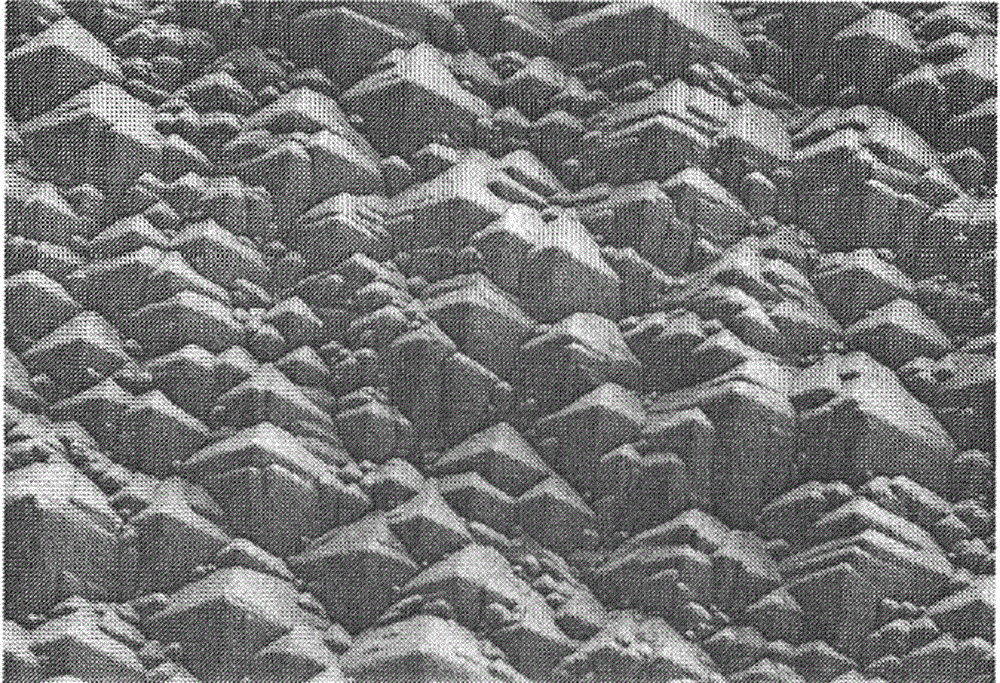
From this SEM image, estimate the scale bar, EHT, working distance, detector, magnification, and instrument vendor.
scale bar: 20000 nm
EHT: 5 kV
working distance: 9.3 mm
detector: SE(M)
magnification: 2 K X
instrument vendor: Hitachi